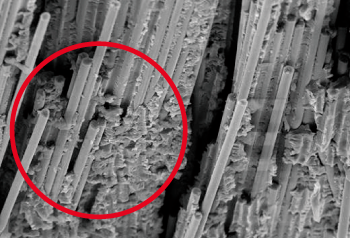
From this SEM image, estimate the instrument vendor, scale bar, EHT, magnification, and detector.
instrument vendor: JEOL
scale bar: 50000 nm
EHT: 15 kV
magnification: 0.5 K X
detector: SEI